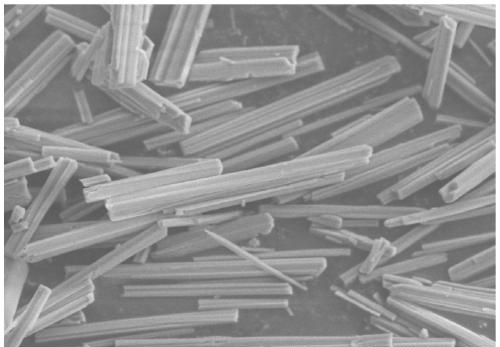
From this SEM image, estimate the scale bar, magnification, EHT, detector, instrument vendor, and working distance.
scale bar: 10000 nm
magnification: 5 K X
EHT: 5 kV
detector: SE(M)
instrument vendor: Hitachi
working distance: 9.1 mm